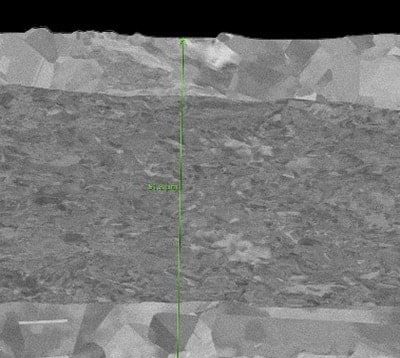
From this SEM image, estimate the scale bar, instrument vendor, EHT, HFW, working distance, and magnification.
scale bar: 10000 nm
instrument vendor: Thermo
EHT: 5 kV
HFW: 59.7 µm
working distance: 7.024 mm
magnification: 4.5 K X